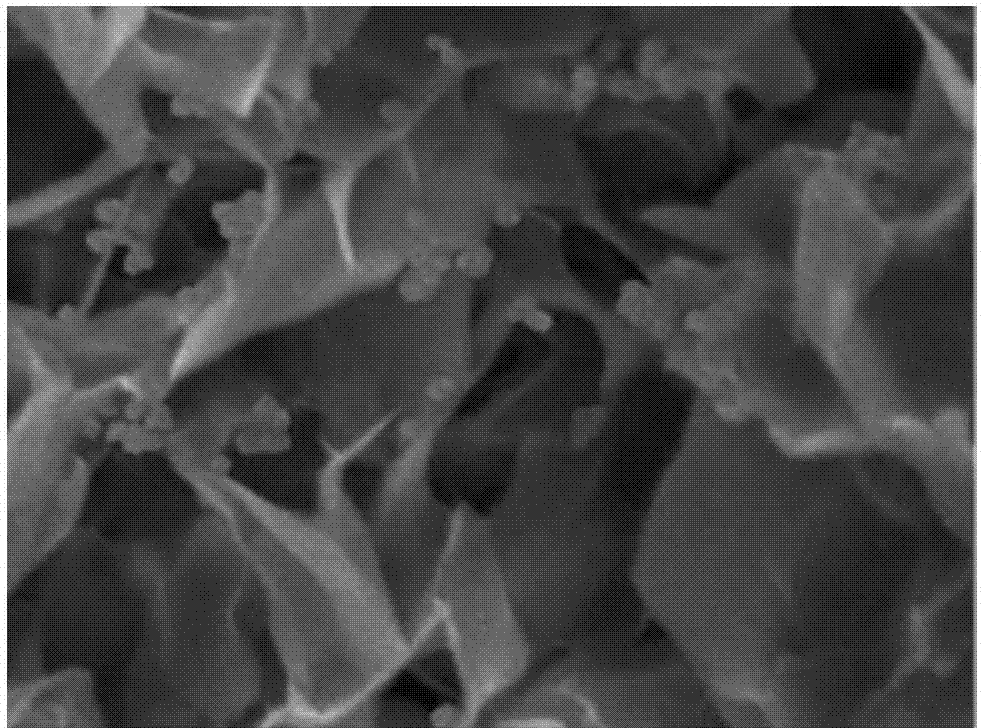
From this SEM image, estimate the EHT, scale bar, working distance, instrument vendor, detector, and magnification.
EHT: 5 kV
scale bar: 100 nm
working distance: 7.9 mm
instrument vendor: JEOL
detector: SEI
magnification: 30 K X